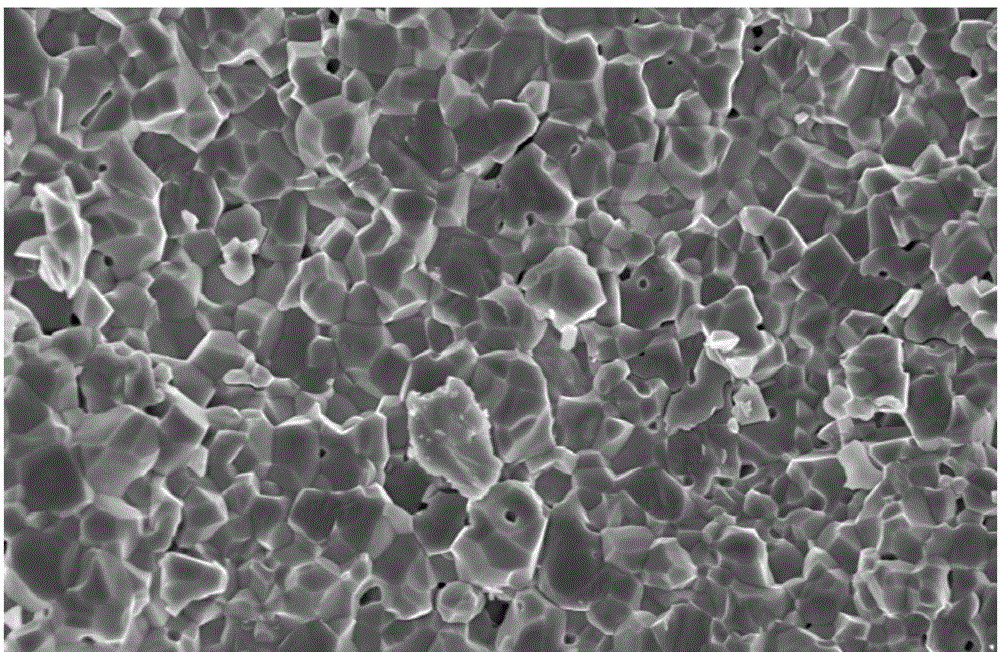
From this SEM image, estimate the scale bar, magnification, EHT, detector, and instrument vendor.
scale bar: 20000 nm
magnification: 2 K X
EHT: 15 kV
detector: SE(M)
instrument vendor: Hitachi